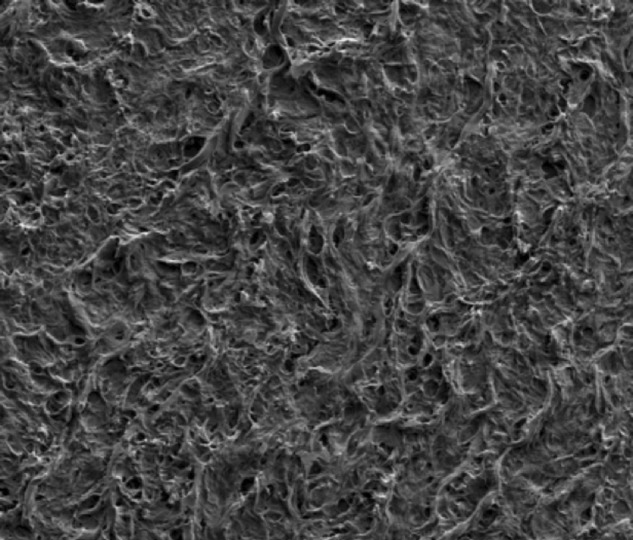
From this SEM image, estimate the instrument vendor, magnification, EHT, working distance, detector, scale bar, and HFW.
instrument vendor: FEI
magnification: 1.5 K X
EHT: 5 kV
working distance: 7.8 mm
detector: LVSED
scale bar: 50000 nm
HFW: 199 µm